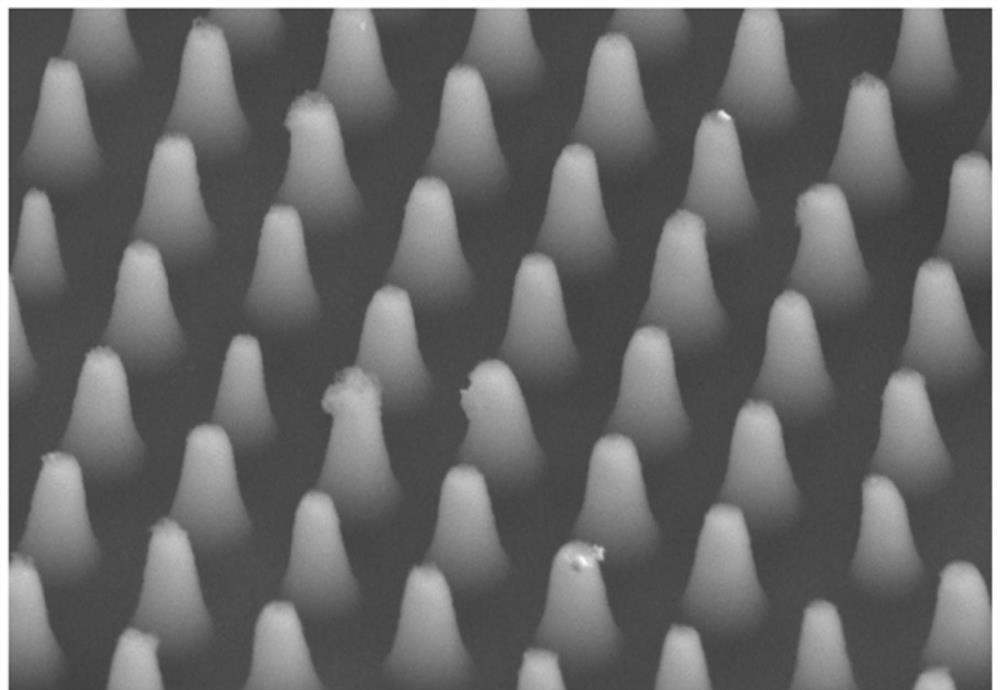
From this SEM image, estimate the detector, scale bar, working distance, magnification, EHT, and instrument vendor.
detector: LA10(UL)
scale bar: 2000 nm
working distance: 12.4 mm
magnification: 22 K X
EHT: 5 kV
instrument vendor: Hitachi